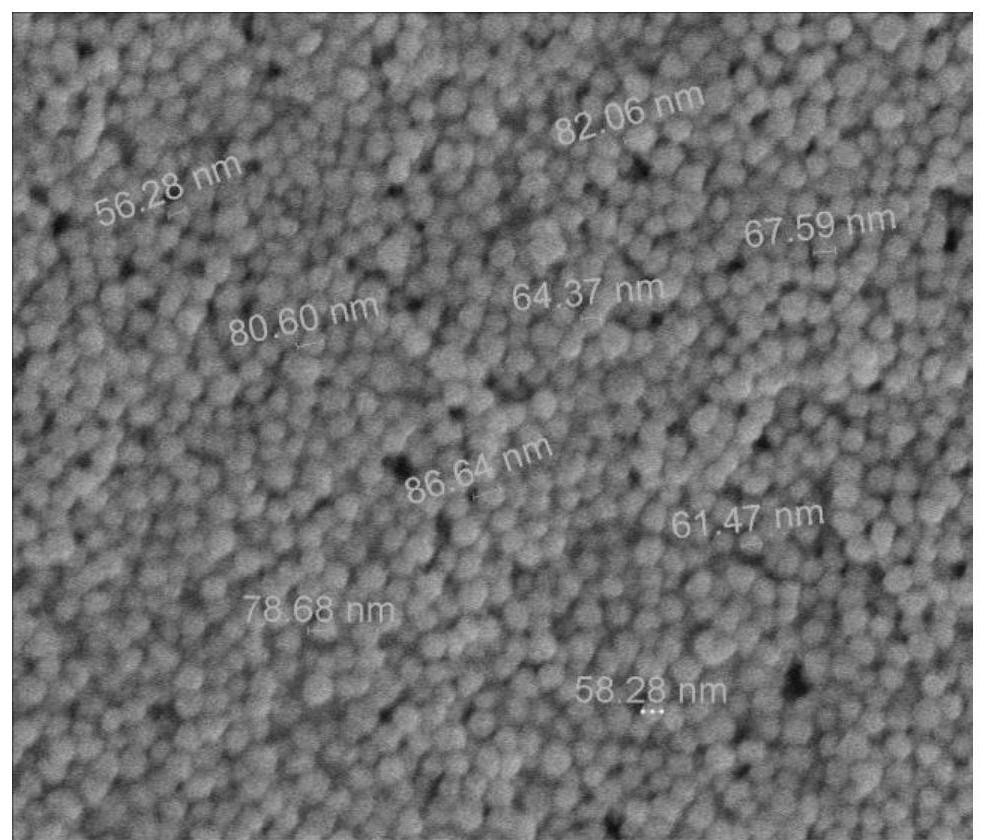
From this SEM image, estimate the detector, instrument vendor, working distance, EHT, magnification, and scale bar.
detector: TLD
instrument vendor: FEI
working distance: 4.5 mm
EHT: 5 kV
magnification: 50 K X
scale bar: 500 nm